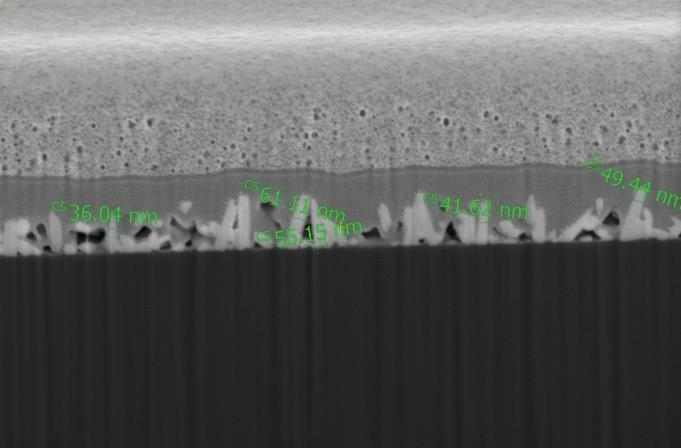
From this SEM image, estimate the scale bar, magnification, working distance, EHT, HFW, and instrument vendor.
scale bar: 1000 nm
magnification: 150 K X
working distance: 3.9 mm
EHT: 2 kV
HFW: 2.76 µm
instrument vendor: FEI